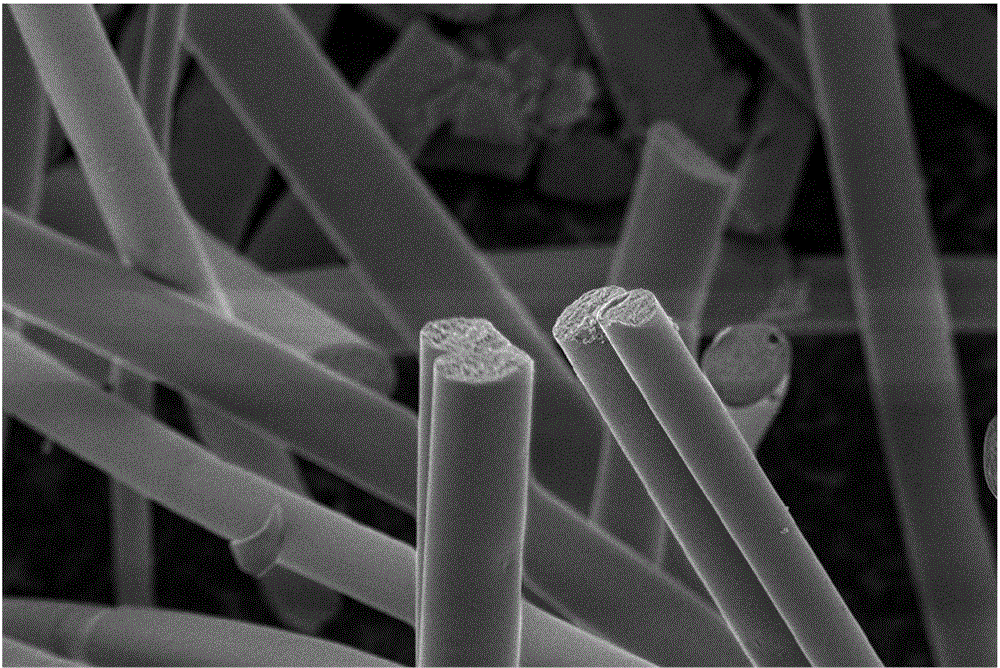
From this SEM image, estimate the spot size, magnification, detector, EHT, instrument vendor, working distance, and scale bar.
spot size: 2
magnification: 4 K X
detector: ETD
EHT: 3 kV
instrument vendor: FEI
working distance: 9.2 mm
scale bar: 40000 nm